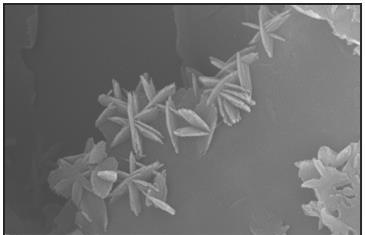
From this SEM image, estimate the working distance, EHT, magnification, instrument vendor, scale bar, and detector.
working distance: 6 mm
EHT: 25 kV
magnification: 44.3 K X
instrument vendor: Zeiss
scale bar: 200 nm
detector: SE2